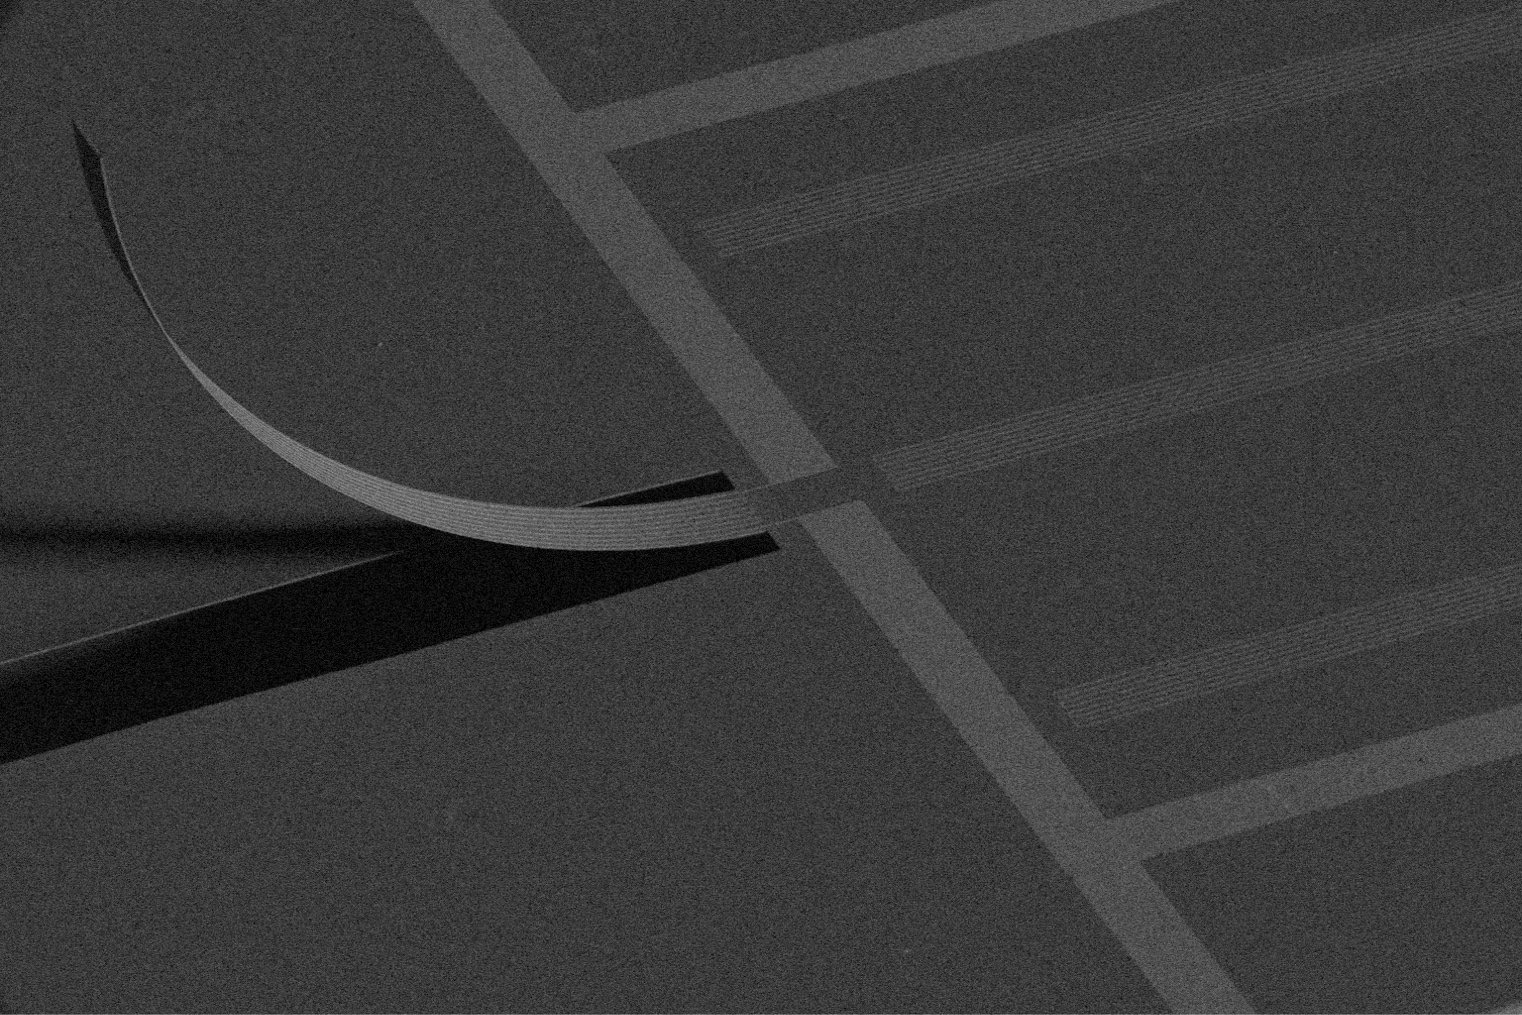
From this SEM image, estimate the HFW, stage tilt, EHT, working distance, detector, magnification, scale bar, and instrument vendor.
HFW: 2070 µm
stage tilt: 52°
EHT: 5 kV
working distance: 3.8 mm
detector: ETD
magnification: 0.2 K X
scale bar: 500000 nm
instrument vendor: FEI